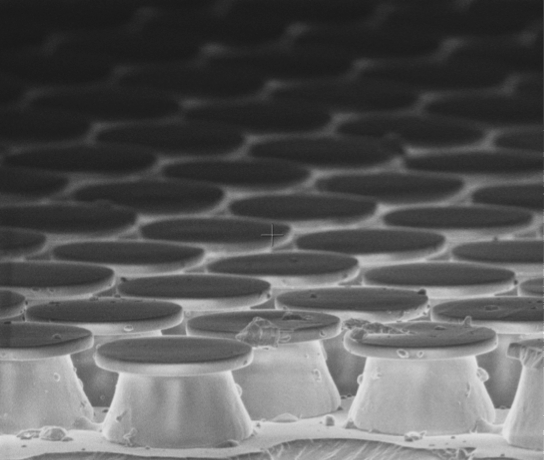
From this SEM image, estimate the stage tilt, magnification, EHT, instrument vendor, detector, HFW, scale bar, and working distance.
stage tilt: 10°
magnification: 5 K X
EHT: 2 kV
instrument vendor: FEI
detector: TLD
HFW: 59.7 µm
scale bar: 10000 nm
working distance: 5.8 mm